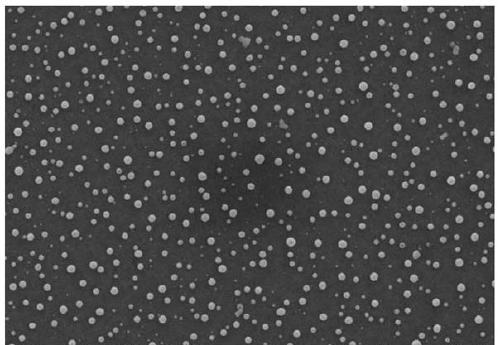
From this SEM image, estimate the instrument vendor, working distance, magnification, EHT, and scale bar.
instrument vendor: Zeiss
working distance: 6 mm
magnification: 5 K X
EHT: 15 kV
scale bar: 1000 nm